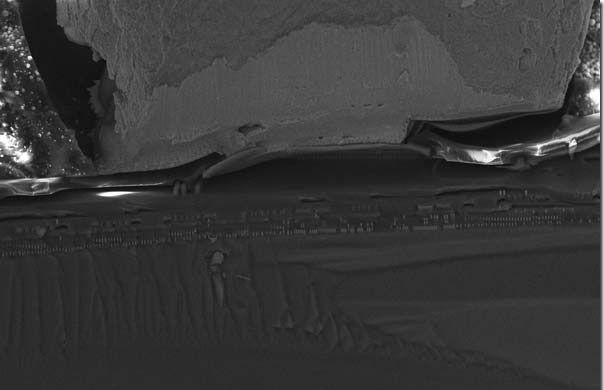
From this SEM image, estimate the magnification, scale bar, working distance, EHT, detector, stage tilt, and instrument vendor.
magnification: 2 K X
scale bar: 10000 nm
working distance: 4 mm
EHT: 15 kV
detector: InLens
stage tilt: -15°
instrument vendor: Zeiss